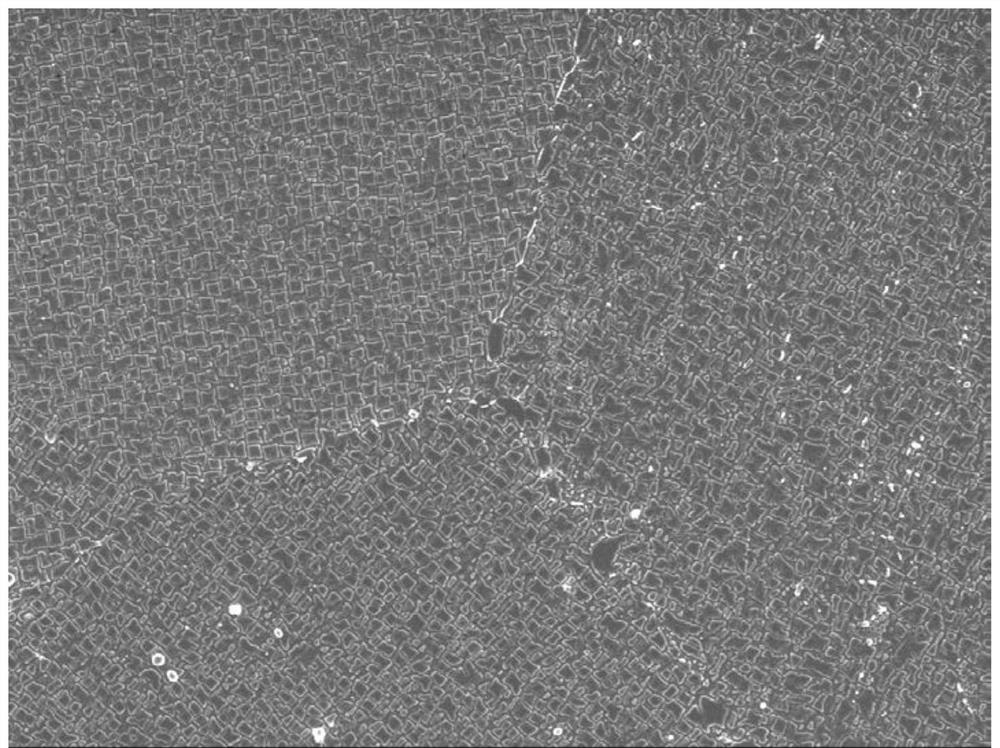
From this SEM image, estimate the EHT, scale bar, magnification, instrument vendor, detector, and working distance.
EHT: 5 kV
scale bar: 1000 nm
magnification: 5 K X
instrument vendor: JEOL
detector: SEI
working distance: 7.3 mm